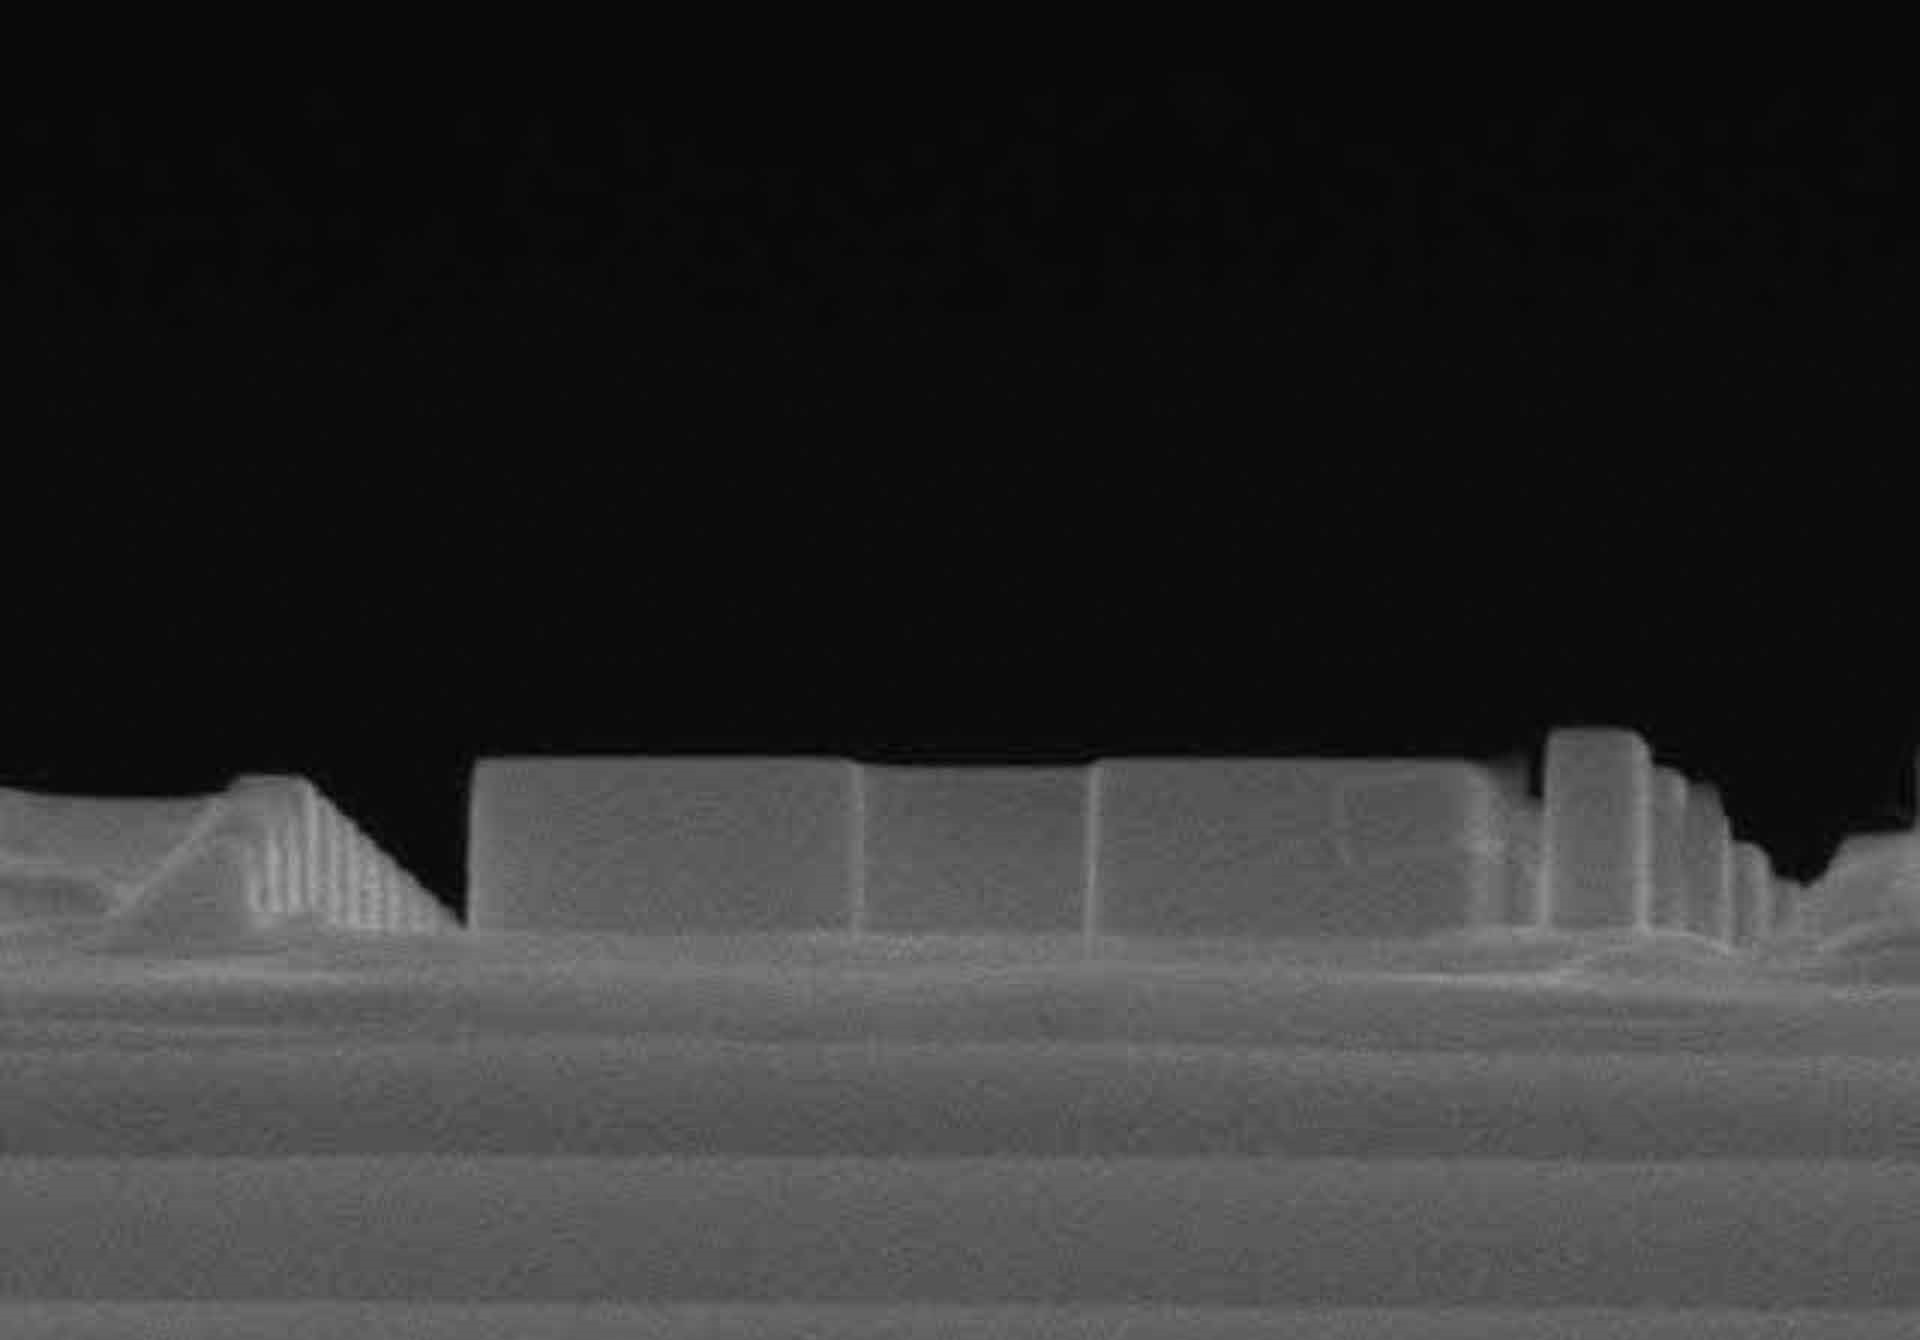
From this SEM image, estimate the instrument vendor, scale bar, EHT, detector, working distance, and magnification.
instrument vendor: Hitachi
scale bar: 1000 nm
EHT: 15 kV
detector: SE(M)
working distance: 15.8 mm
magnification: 45 K X